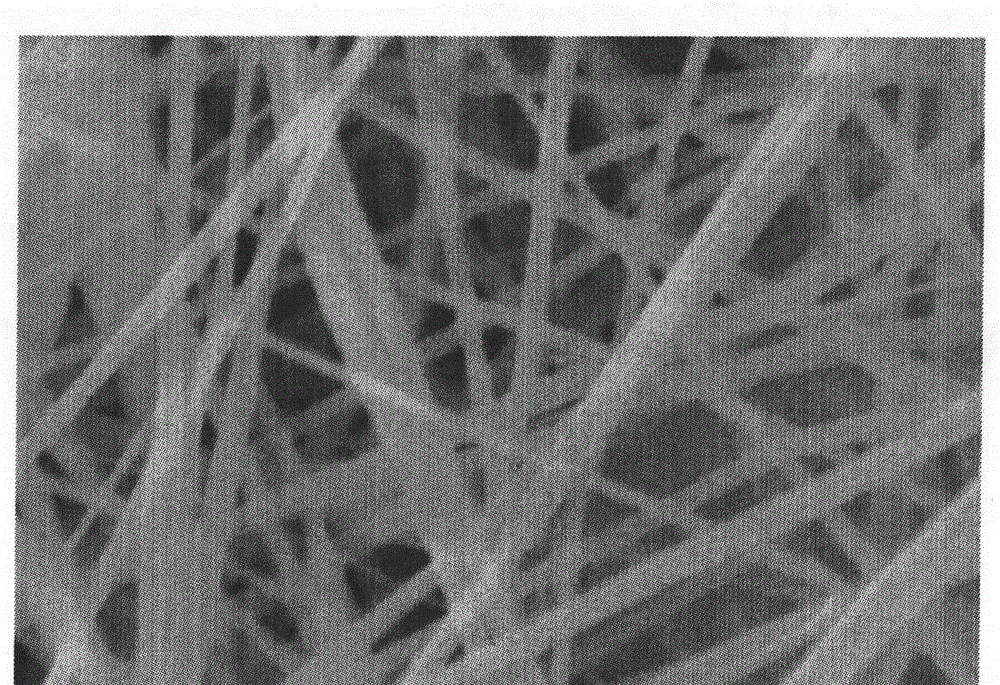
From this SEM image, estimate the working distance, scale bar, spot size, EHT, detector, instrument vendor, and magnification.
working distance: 10 mm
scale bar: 2000 nm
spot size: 4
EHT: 20 kV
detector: SE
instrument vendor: FEI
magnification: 20 K X